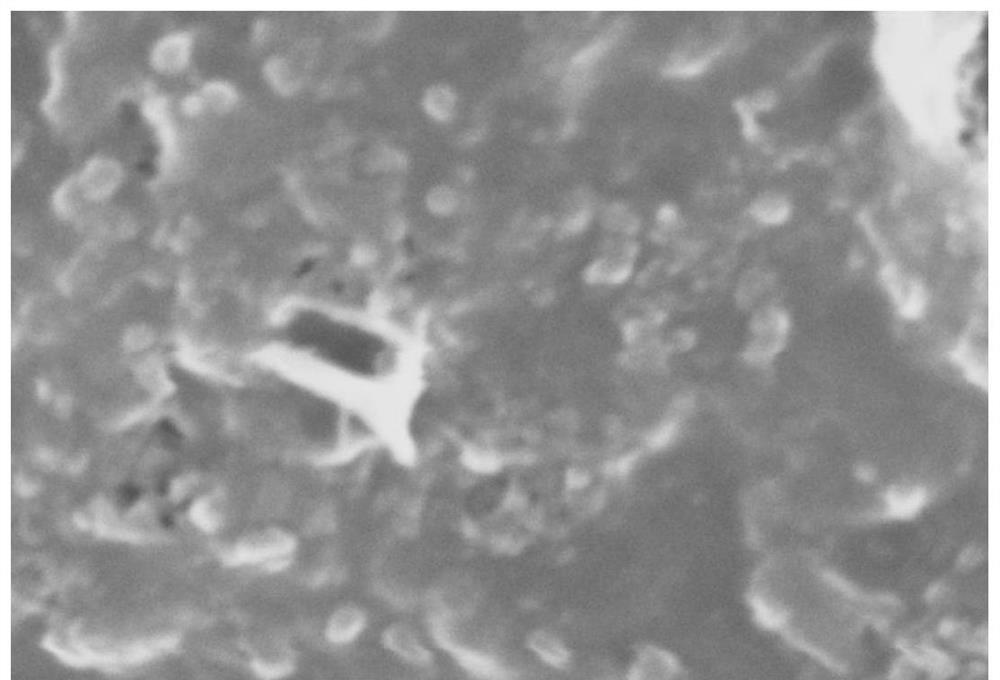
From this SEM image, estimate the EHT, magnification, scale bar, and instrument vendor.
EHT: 20 kV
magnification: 10 K X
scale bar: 1000 nm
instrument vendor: other